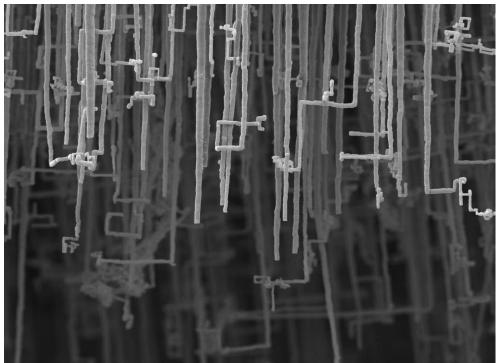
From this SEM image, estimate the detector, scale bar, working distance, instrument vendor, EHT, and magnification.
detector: LED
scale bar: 1000 nm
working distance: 10 mm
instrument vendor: JEOL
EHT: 5 kV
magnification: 4 K X